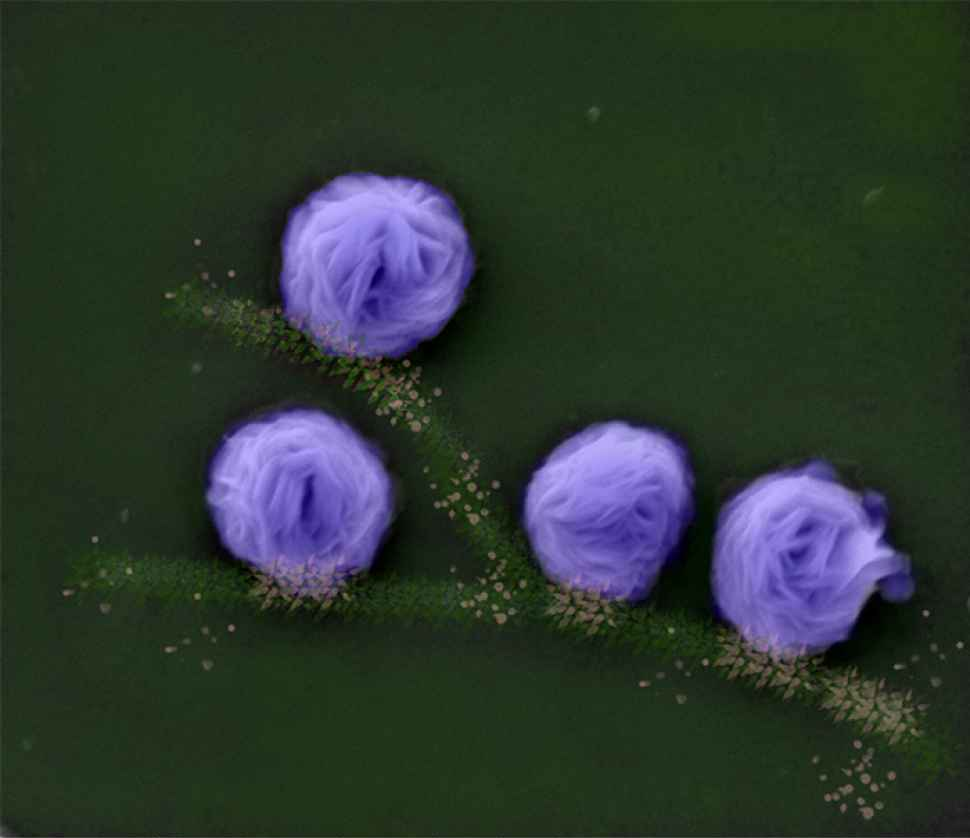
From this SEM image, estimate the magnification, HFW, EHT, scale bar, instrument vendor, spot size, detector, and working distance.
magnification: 24 K X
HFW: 12.4 µm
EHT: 10 kV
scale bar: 5000 nm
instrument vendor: FEI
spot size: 4.5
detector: ETD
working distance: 9.5 mm